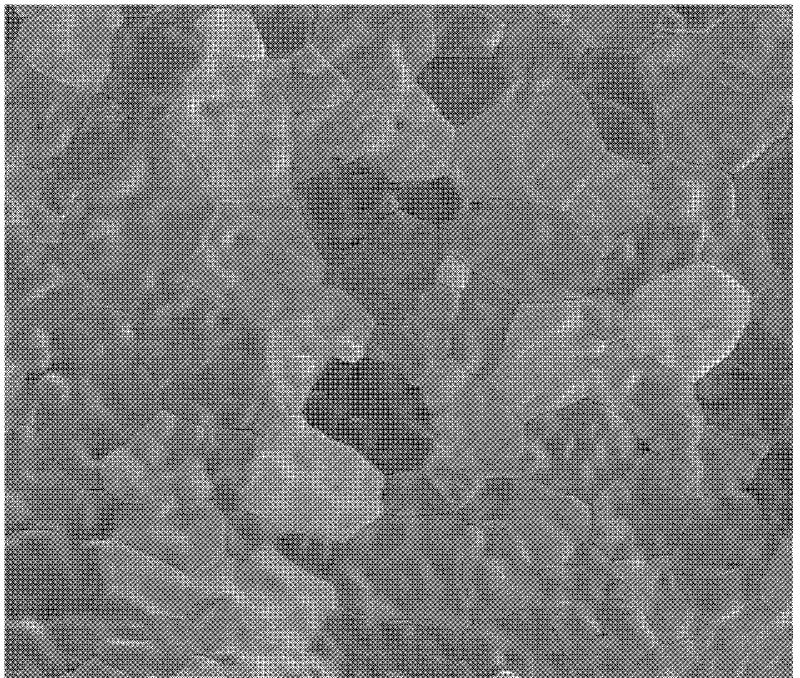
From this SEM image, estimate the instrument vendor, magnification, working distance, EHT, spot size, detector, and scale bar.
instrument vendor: FEI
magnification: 10 K X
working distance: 6 mm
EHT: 5 kV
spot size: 3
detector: vCD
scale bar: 10000 nm